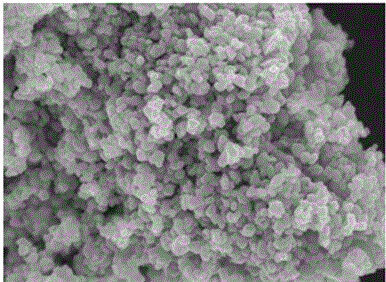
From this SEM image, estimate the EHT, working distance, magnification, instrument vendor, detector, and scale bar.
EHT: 10 kV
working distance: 7.9 mm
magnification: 20 K X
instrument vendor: JEOL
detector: SEI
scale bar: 1000 nm